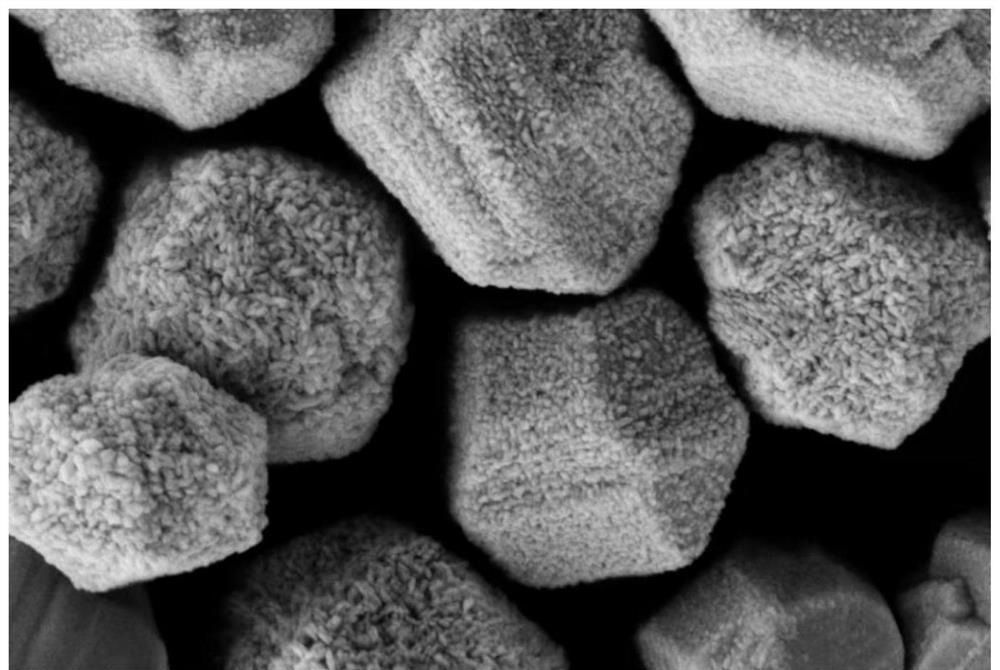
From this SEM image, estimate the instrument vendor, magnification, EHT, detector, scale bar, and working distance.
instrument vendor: Zeiss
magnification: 30 K X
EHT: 5 kV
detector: SE2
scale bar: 200 nm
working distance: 8.3 mm